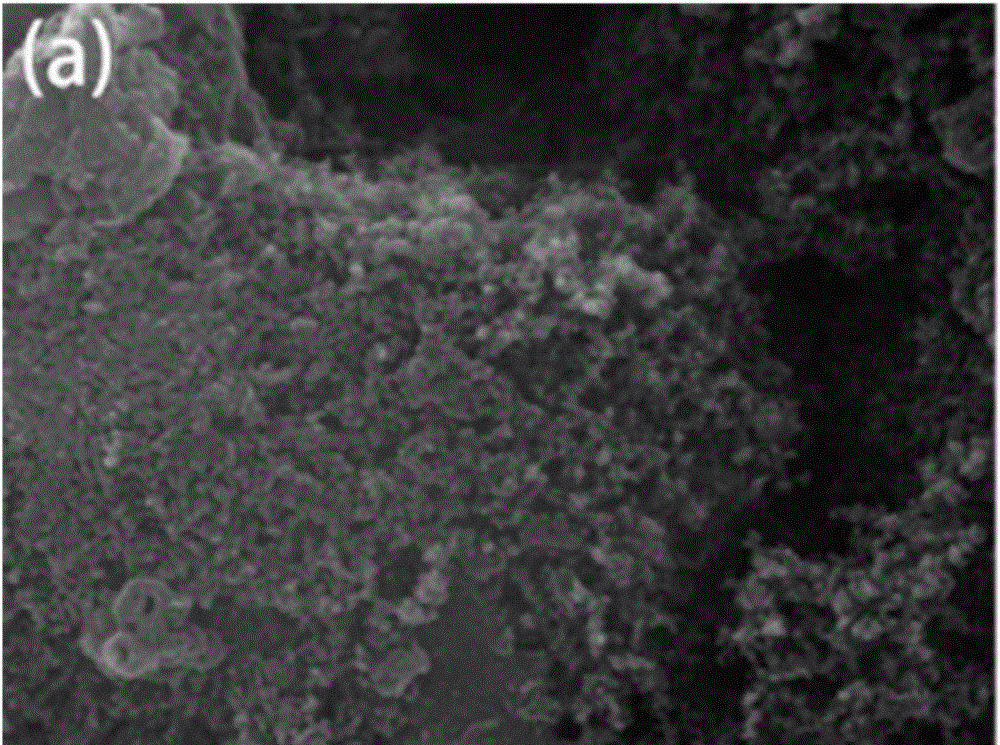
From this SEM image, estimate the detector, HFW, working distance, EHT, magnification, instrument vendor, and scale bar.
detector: SE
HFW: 10.4 µm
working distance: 8 mm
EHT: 30 kV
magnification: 20 K X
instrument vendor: Tescan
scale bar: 2000 nm